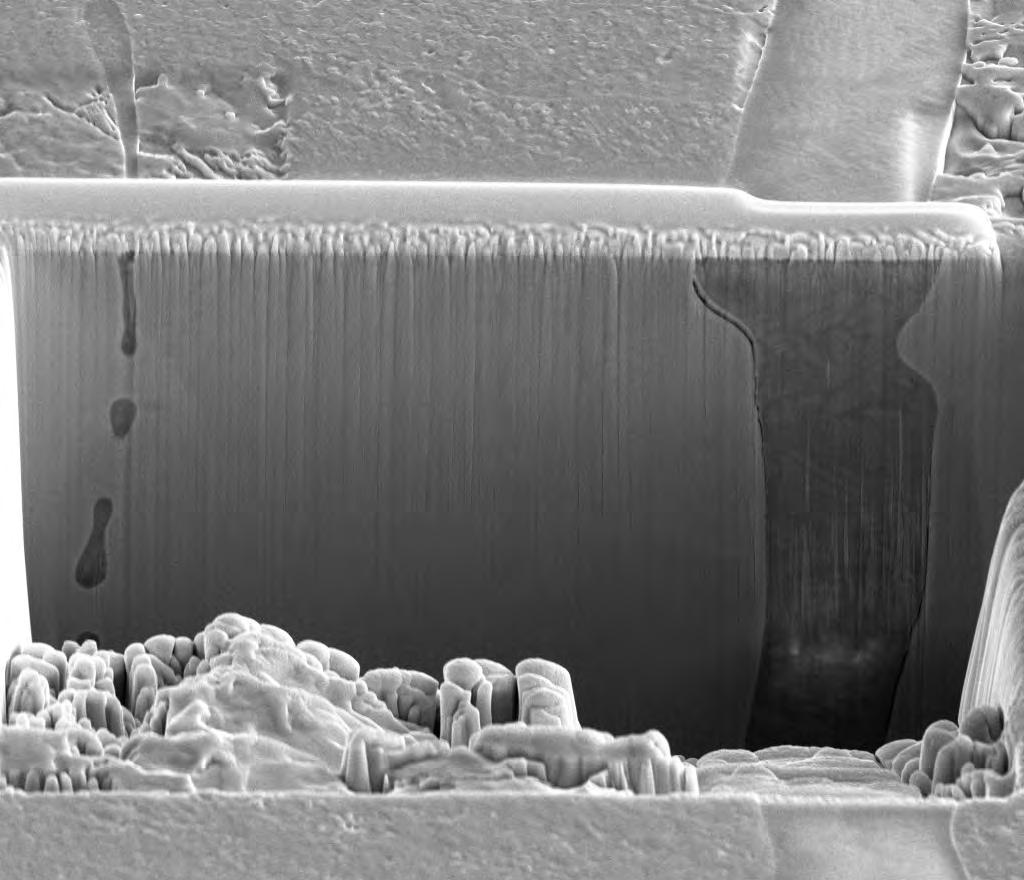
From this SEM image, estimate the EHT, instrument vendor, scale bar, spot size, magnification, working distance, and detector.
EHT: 10 kV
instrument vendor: FEI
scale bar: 10000 nm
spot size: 4.5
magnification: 5 K X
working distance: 10 mm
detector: ETD